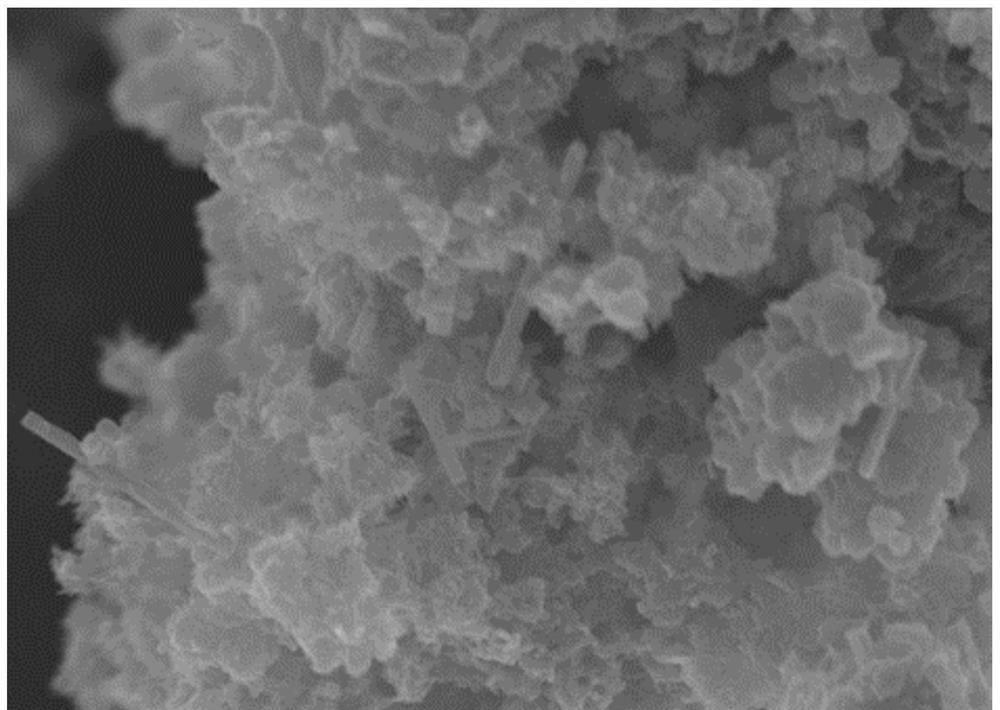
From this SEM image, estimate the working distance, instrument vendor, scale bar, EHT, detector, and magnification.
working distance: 3.9 mm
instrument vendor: Hitachi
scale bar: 2000 nm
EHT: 10 kV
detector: SE(UL)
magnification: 25 K X